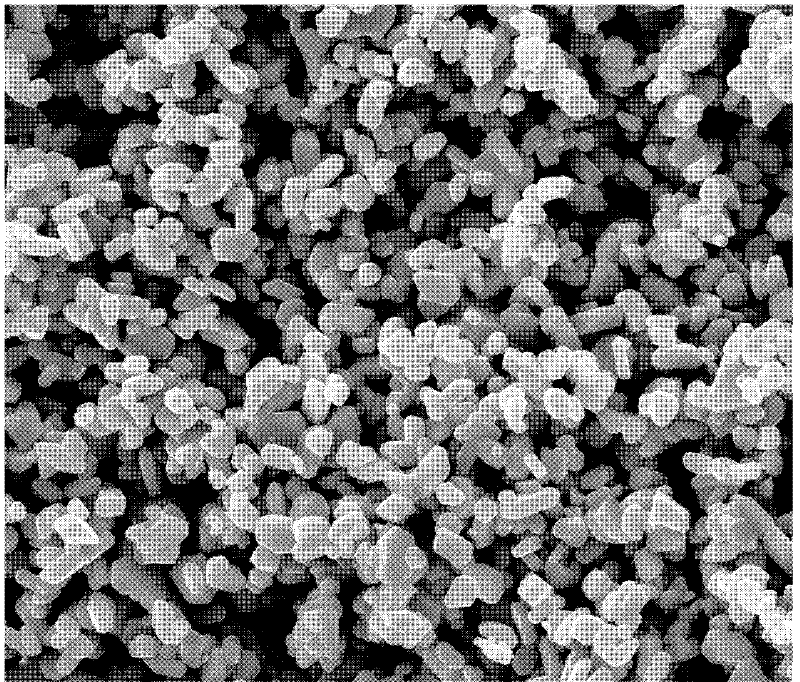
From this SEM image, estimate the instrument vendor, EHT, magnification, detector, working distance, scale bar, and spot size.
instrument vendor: FEI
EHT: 20 kV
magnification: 10 K X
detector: ETD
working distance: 11.5 mm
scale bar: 5000 nm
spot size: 3.5